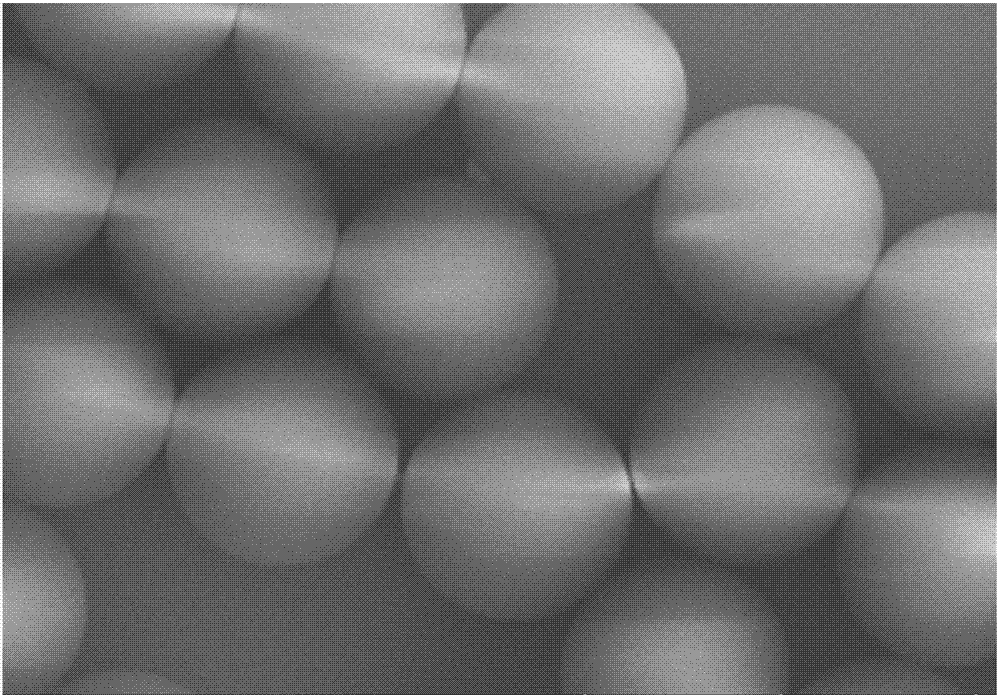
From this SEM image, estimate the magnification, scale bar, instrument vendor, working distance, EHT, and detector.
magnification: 10 K X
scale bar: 5000 nm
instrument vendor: Hitachi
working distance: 13.6 mm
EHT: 10 kV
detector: SE(UL)